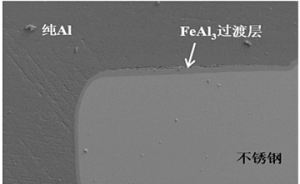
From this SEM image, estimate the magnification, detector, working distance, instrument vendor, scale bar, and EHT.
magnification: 0.06 K X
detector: SE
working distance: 13.7 mm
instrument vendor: Hitachi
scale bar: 500000 nm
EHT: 20 kV